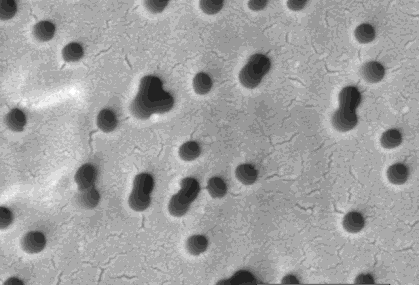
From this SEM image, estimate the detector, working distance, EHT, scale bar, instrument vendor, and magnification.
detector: SE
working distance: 8.1 mm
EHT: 5 kV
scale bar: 1000 nm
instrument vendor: JEOL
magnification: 40 K X